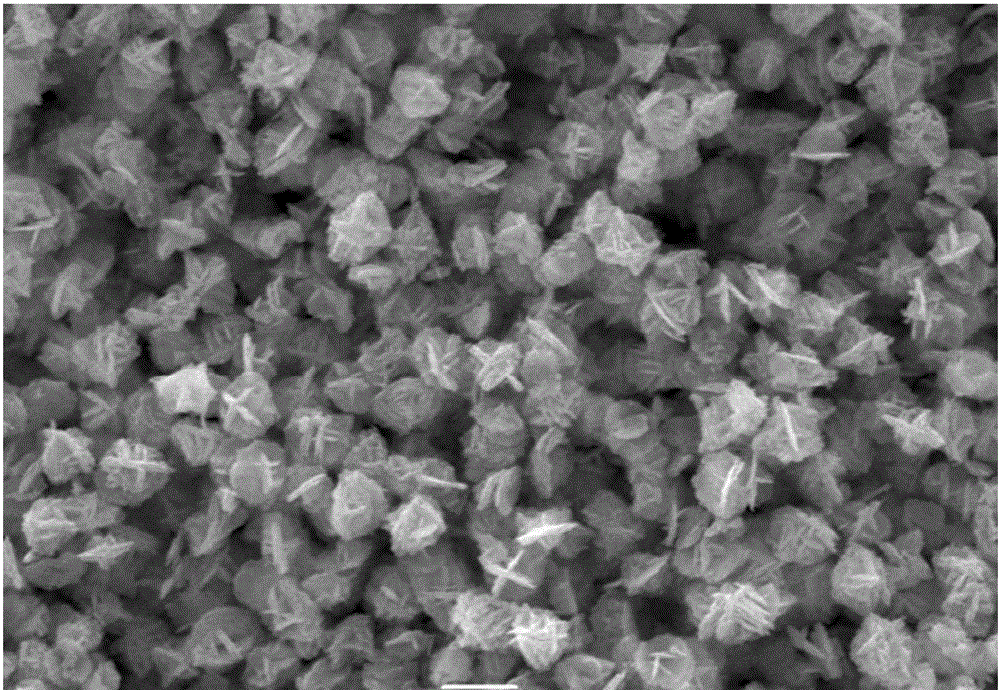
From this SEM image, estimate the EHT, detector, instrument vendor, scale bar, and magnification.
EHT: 30 kV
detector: SEI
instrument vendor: JEOL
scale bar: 1000 nm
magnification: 10 K X